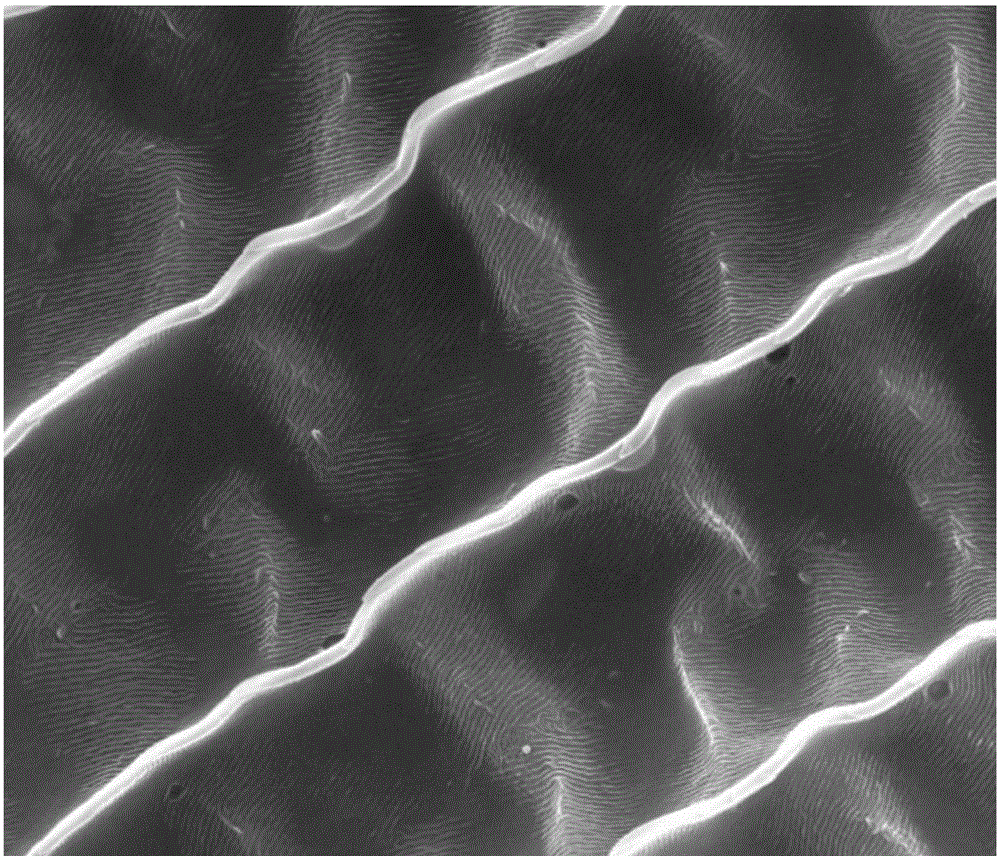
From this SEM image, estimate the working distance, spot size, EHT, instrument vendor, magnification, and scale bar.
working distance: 9.2 mm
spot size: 3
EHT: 20 kV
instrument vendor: FEI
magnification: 12 K X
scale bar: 10000 nm